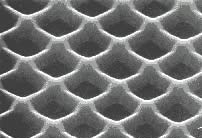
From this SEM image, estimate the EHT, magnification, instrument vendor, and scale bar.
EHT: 30 kV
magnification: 60 K X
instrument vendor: JEOL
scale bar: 500 nm